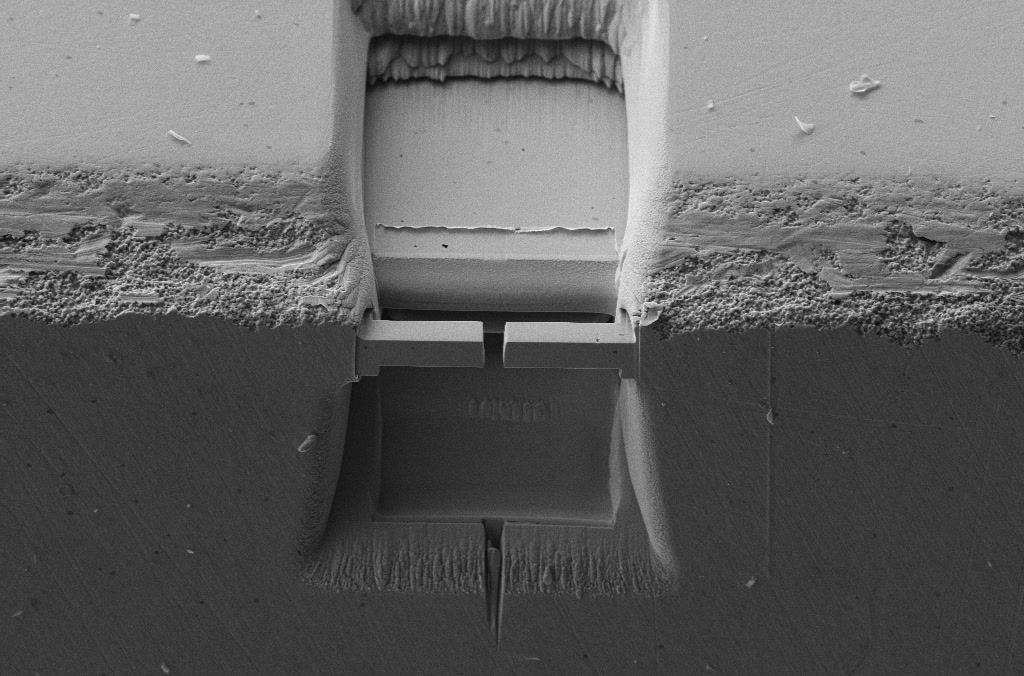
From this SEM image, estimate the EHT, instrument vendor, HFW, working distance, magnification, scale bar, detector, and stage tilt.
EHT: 5 kV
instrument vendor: Zeiss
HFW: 90.67 µm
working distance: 4.8 mm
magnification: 3.31 K X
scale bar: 10000 nm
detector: SE2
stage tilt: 36°